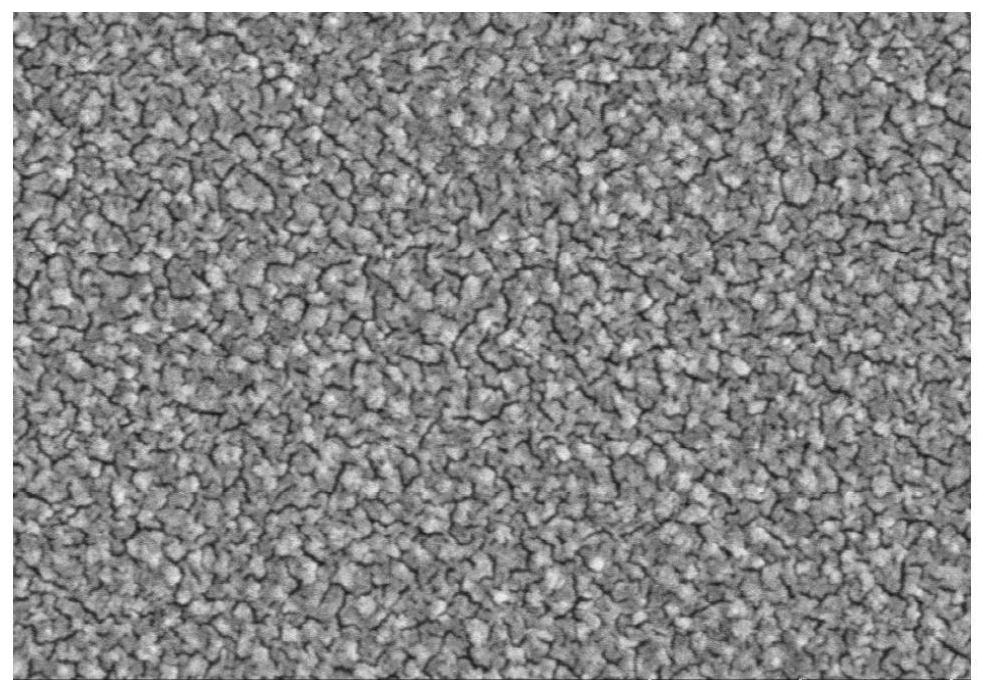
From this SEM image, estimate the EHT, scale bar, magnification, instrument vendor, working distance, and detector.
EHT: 5 kV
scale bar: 500 nm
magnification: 80 K X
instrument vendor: Hitachi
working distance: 10.8 mm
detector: SE(TUL)